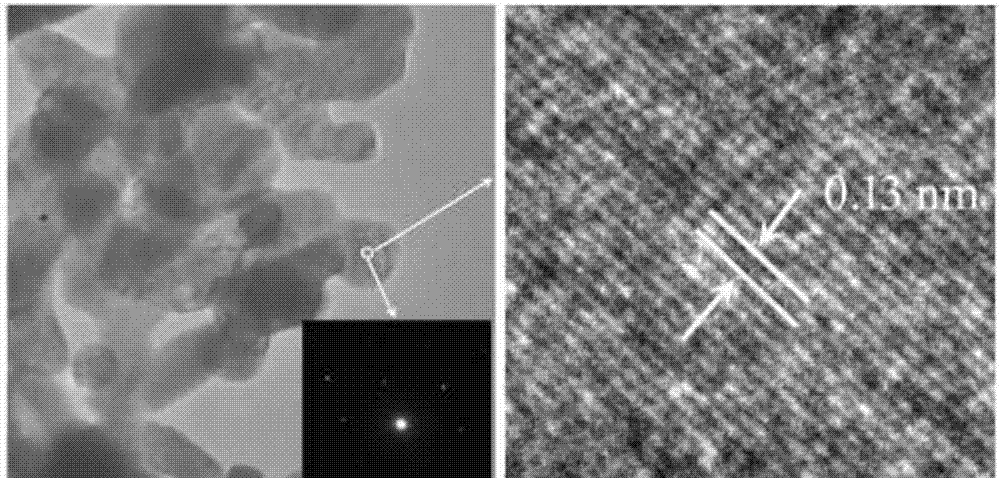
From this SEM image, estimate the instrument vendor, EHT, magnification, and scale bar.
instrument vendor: JEOL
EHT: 200 kV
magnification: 100 K X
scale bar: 200 nm